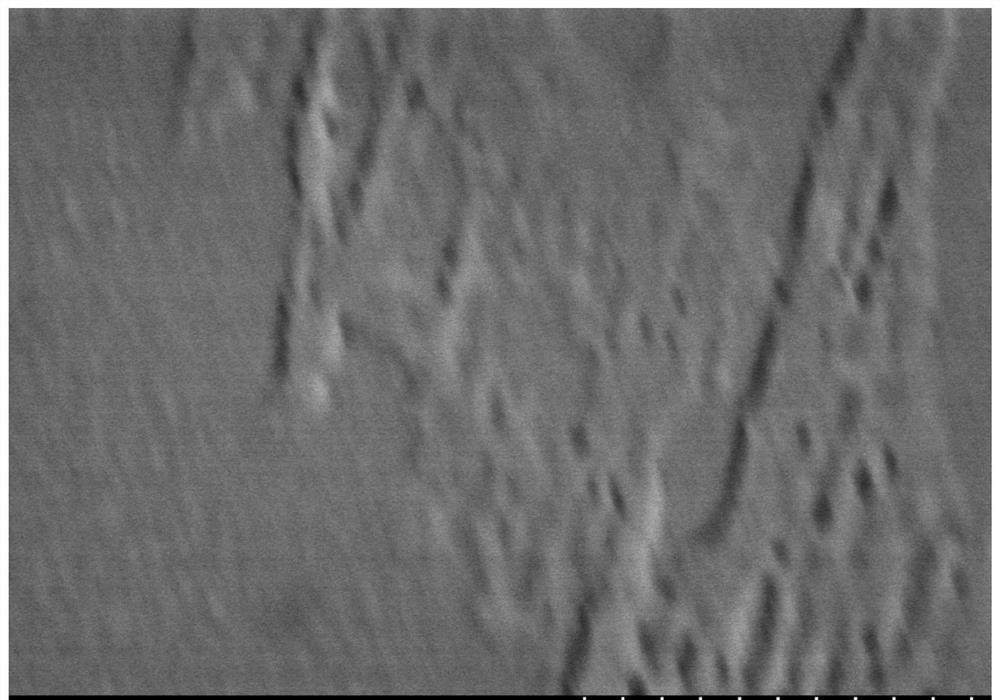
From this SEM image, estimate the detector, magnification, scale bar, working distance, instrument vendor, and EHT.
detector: SE(M)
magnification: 100 K X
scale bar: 500 nm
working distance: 9 mm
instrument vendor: Hitachi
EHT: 3 kV